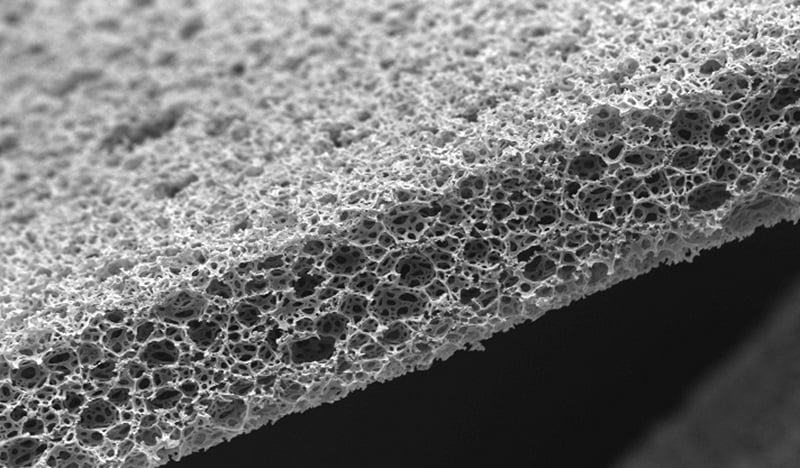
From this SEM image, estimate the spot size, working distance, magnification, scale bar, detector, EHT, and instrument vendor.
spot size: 5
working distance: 9.6 mm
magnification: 0.163 K X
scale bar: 200000 nm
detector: SE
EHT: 15 kV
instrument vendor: FEI